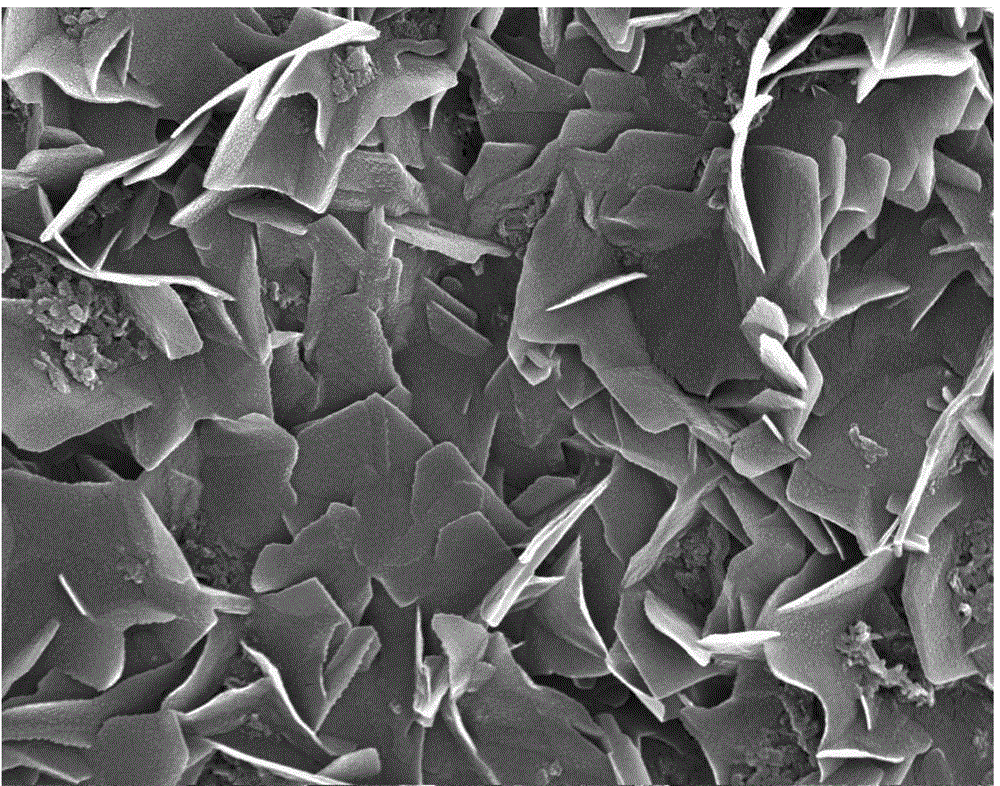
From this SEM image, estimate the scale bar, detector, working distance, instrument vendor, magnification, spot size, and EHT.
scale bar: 3000 nm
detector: TLD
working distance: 5.2 mm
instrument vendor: FEI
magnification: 20 K X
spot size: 3.5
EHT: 15 kV